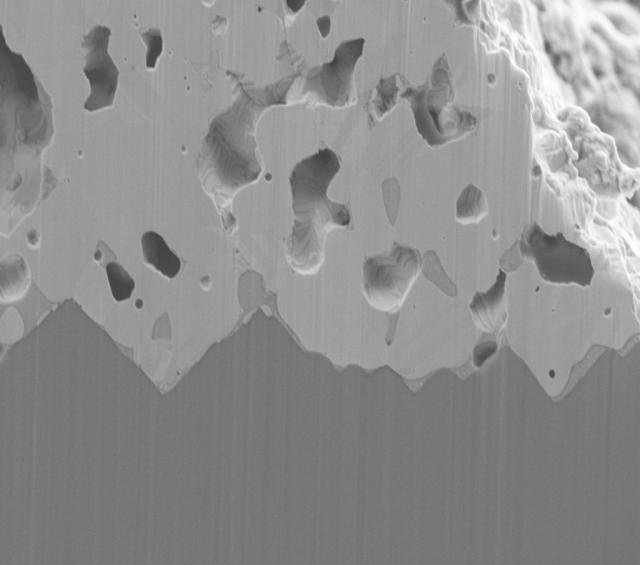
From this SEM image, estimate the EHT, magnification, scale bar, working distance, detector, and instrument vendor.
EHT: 4 kV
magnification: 5 K X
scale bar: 2000 nm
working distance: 3.9 mm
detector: SE2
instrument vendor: Zeiss